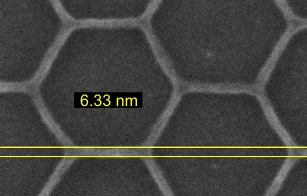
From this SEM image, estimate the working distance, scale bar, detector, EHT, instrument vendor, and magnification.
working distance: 3 mm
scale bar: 20 nm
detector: InLens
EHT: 8 kV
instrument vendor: Zeiss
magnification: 500 K X